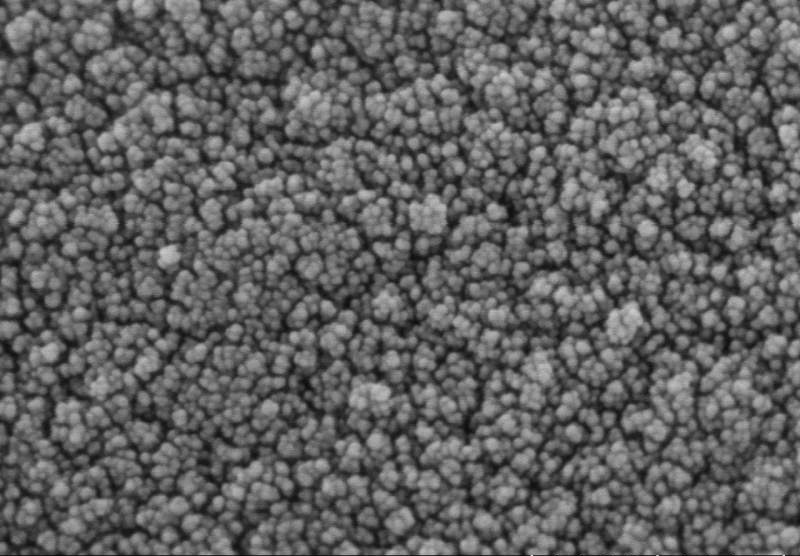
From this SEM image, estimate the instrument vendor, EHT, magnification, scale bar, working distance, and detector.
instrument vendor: Hitachi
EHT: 3 kV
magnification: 200 K X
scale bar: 200 nm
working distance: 3.3 mm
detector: SE(UL)